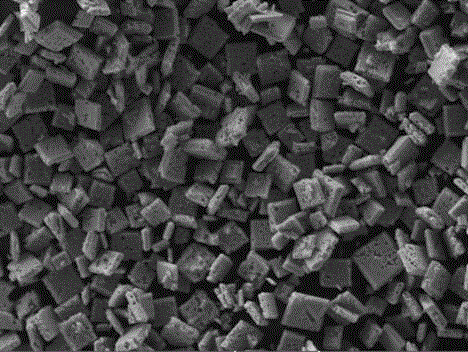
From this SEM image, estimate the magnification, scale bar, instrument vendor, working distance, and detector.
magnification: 0.5 K X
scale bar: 50000 nm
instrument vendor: Tescan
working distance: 7.66 mm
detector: SE